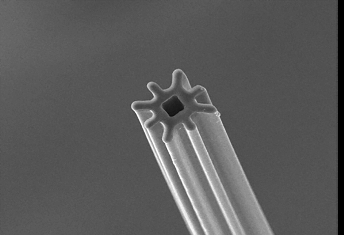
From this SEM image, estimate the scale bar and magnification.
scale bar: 10000 nm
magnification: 1 K X